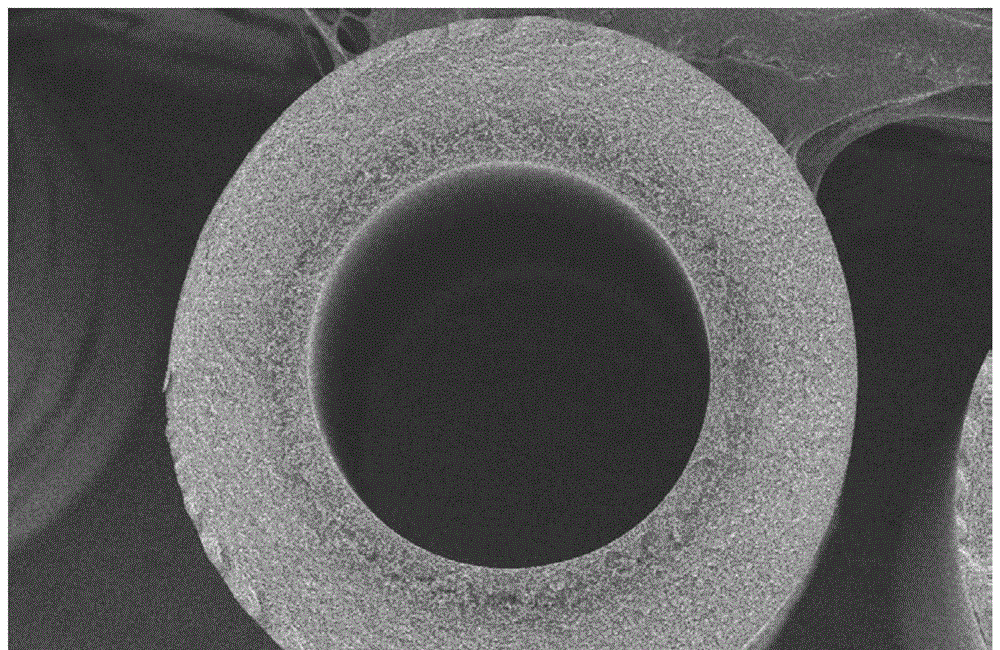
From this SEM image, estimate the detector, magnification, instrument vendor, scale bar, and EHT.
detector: SEI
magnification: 0.095 K X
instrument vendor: JEOL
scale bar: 200000 nm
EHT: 5 kV